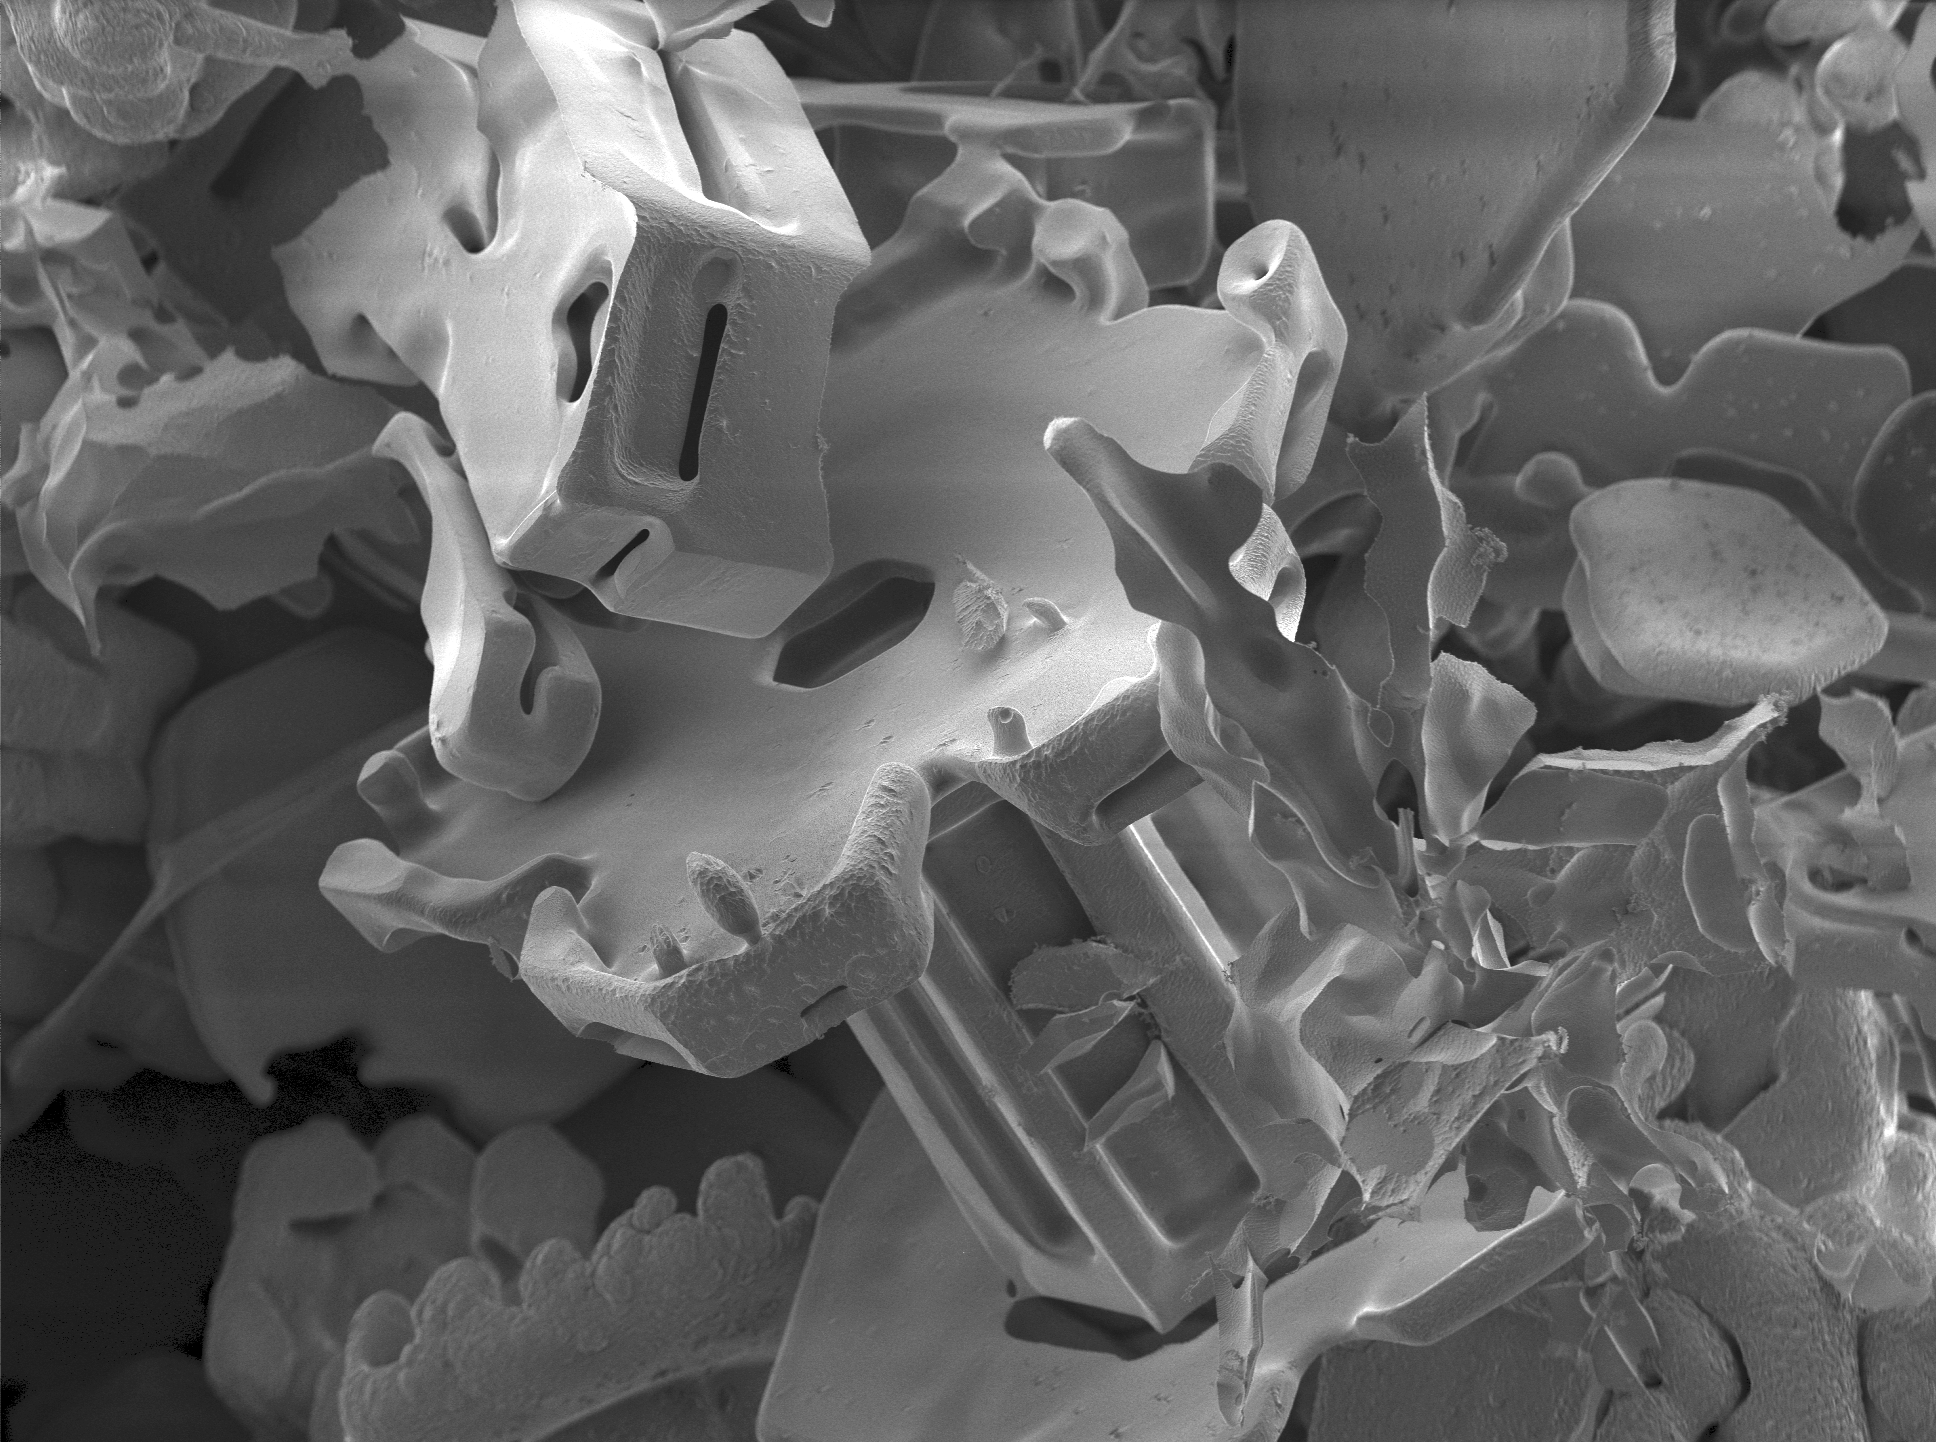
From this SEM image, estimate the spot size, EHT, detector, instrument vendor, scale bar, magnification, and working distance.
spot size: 3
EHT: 2 kV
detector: SE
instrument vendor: FEI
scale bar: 200000 nm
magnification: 0.097 K X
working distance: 10 mm